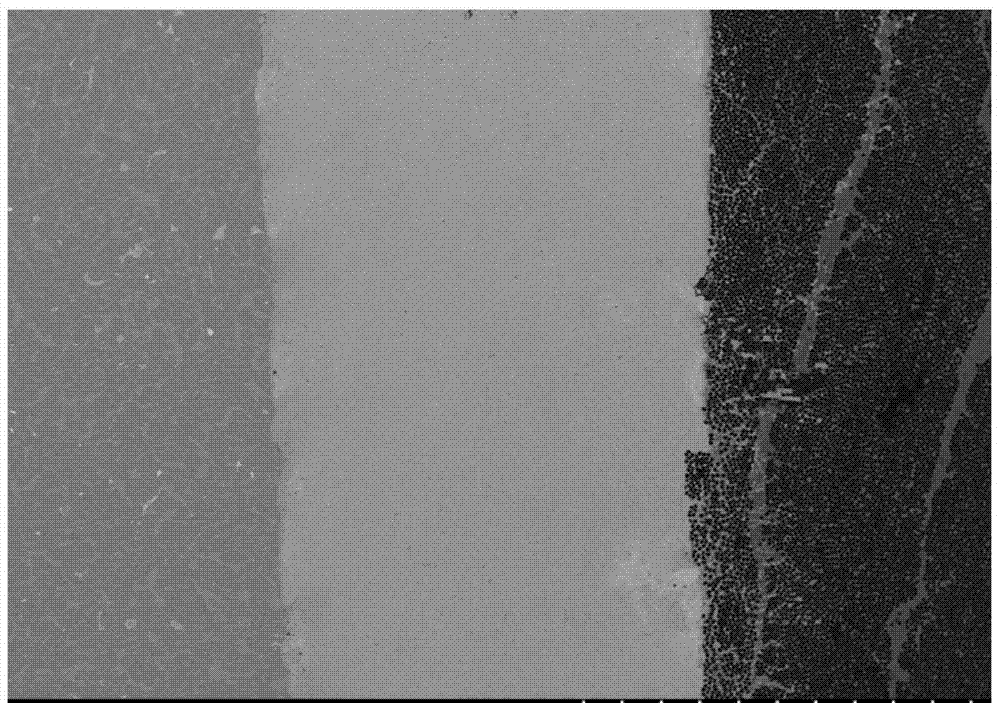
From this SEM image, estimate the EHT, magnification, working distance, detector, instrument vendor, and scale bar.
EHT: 20 kV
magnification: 0.1 K X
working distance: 9.6 mm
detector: BSE3D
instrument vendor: Hitachi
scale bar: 500000 nm